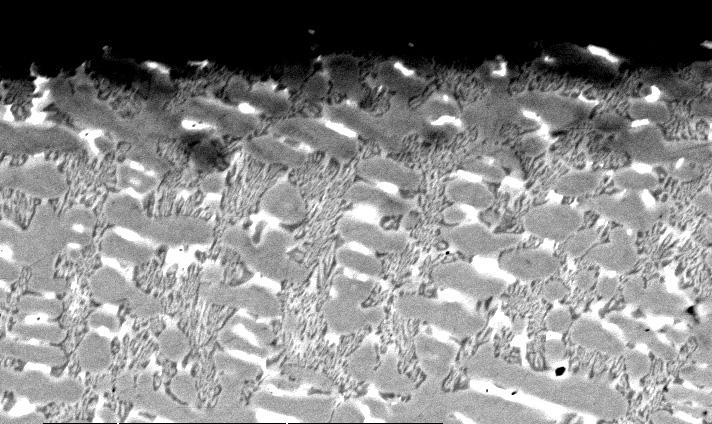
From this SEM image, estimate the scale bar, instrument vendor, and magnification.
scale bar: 20000 nm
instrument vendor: FEI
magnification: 1.4 K X